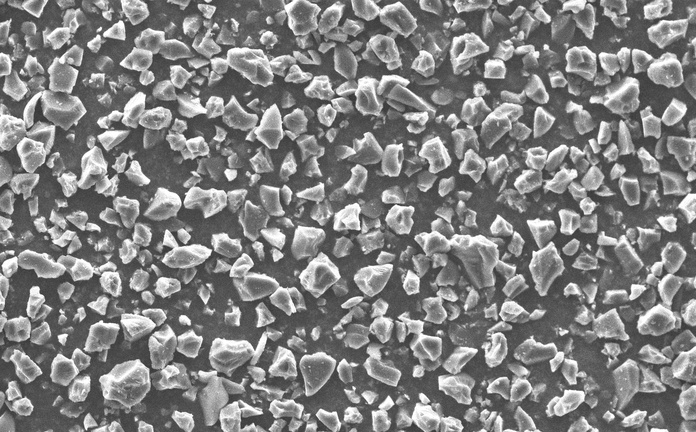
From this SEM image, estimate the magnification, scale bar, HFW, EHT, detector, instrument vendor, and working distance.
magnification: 3 K X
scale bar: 50000 nm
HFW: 173 µm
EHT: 10 kV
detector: SED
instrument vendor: other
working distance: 5.738 mm